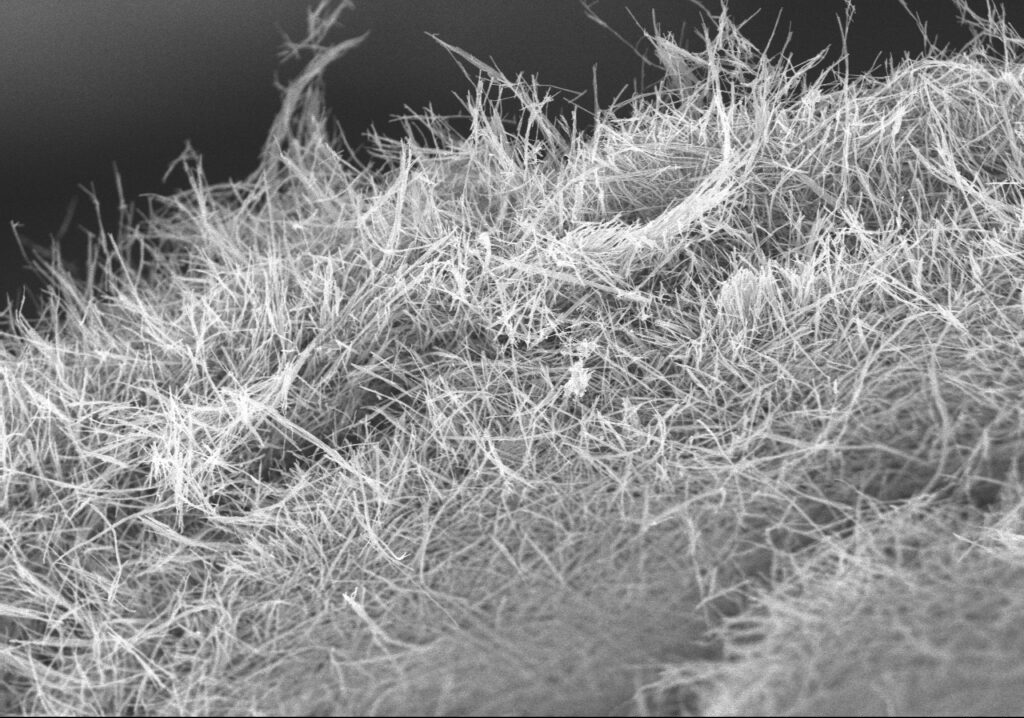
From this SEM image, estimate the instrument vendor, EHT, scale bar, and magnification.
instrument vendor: Hitachi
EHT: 5 kV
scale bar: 30000 nm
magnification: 1.5 K X